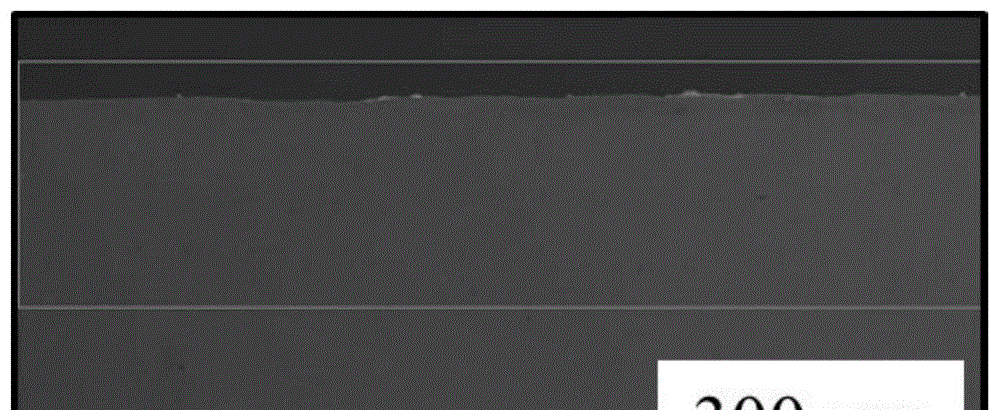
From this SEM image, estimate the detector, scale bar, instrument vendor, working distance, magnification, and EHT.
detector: SE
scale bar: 300000 nm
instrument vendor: Tescan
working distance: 11.9 mm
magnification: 0.1 K X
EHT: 15 kV